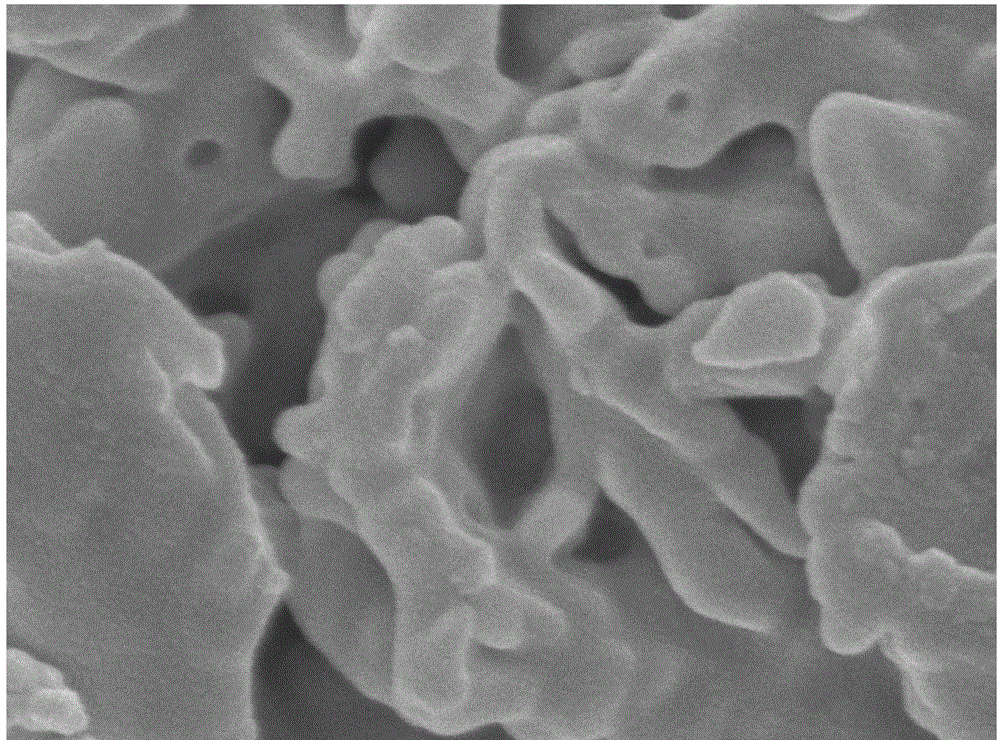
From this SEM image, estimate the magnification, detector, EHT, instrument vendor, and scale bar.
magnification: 100 K X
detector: SEI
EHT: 5 kV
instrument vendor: JEOL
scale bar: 100 nm